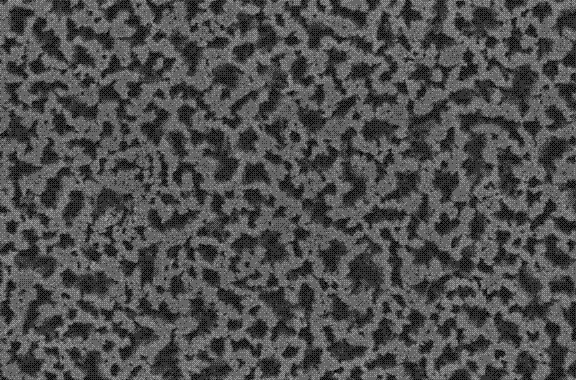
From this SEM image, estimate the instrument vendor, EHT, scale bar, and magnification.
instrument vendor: JEOL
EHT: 20 kV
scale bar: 10000 nm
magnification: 1 K X